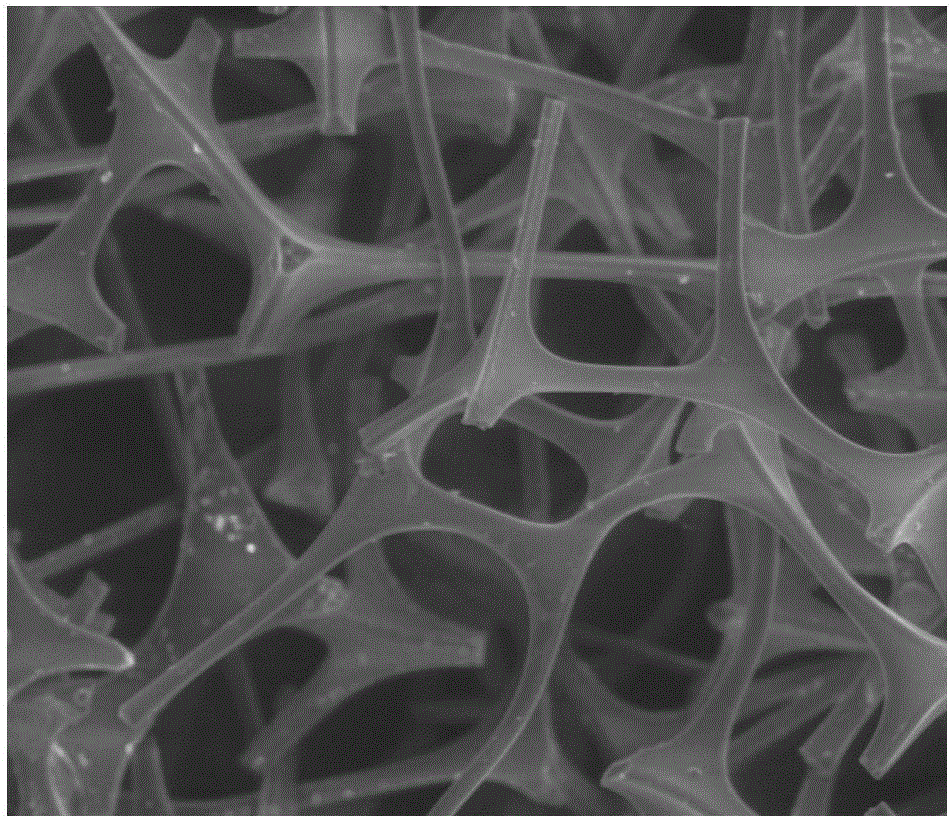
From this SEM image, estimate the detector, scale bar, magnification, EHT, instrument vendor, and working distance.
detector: LFD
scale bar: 50000 nm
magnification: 1.5 K X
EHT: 10 kV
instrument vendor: FEI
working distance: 9.8 mm